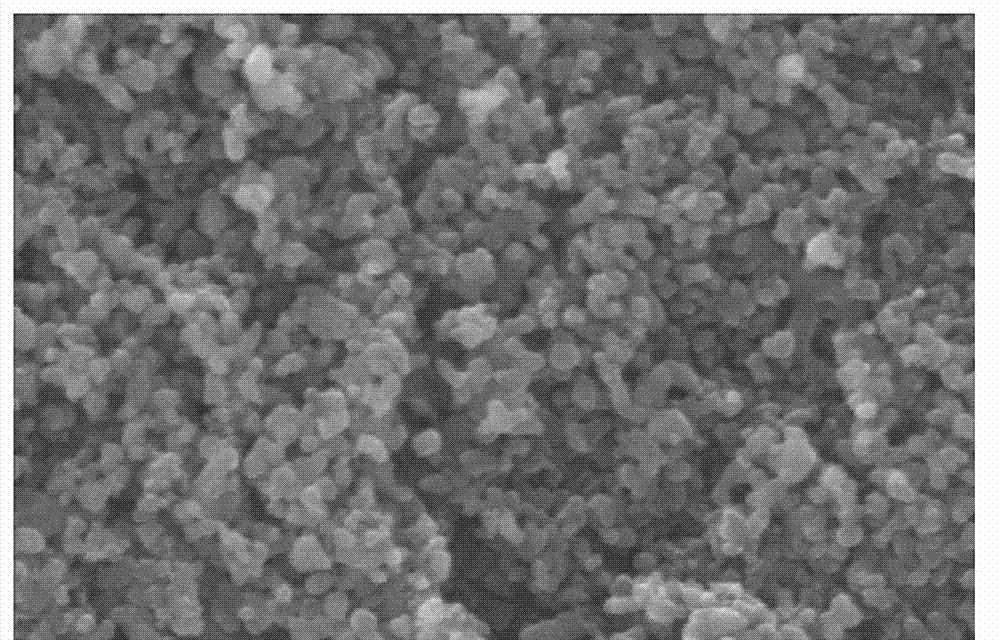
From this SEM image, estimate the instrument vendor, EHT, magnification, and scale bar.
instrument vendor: other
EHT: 25 kV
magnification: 10 K X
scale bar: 1000 nm